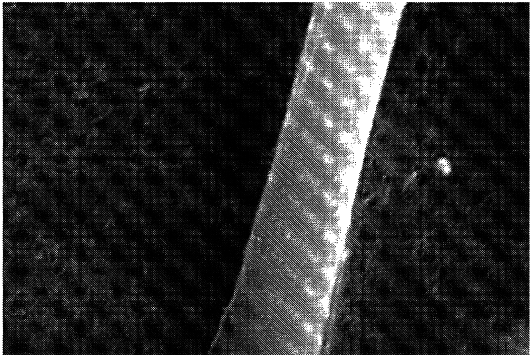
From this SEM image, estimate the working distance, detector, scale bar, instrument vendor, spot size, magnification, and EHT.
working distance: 14 mm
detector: SEI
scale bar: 5000 nm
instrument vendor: JEOL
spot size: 60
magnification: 6 K X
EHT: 20 kV